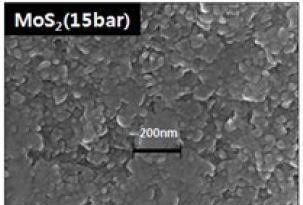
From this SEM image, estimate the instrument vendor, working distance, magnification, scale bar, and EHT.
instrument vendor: Hitachi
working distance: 15 mm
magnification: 100 K X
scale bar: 500 nm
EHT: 15 kV